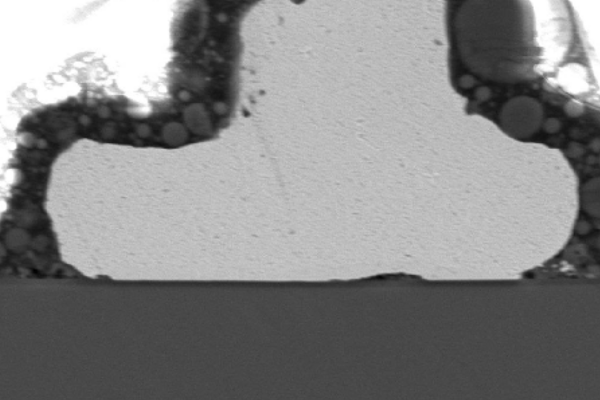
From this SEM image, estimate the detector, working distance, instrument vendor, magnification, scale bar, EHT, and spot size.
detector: SEI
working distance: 35 mm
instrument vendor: JEOL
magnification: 1.7 K X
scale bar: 10000 nm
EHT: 15 kV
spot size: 48